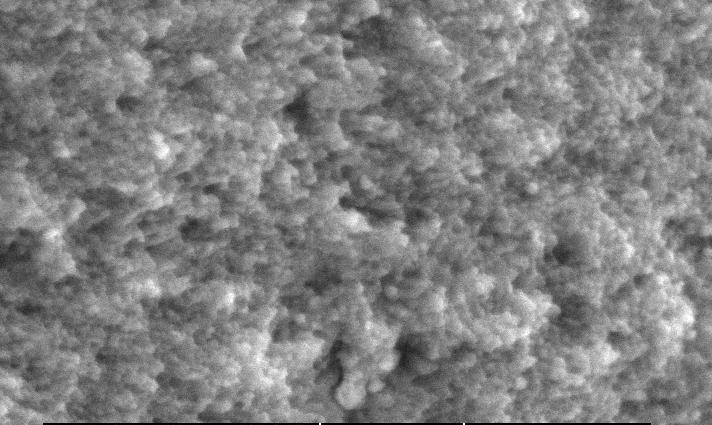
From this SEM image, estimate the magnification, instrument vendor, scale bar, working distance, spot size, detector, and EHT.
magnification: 5 K X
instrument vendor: FEI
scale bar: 5000 nm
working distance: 8.1 mm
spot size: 3.5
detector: SE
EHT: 30 kV